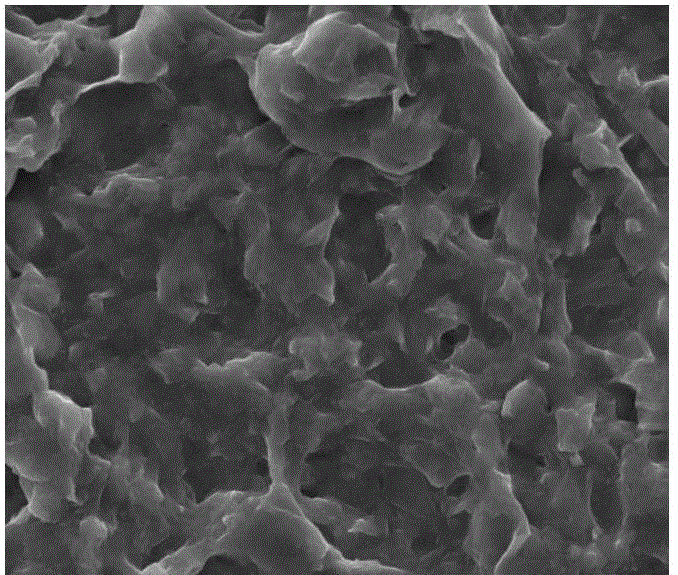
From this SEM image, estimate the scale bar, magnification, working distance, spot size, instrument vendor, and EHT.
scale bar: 10000 nm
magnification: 5 K X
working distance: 10.2 mm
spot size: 4.2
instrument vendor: FEI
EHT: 20 kV